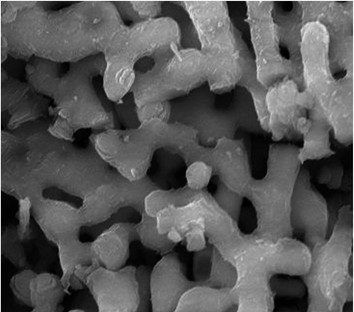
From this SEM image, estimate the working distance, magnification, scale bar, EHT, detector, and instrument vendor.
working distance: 9.7 mm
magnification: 9 K X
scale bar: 5000 nm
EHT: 30 kV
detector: ETD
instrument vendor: FEI